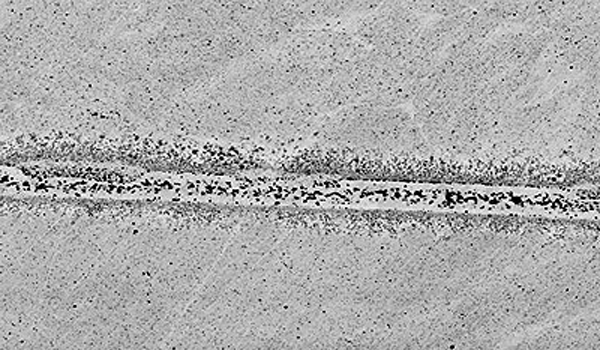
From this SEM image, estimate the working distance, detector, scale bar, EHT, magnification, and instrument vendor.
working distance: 11 mm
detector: COMP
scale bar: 100000 nm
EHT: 15 kV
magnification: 0.25 K X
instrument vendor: JEOL